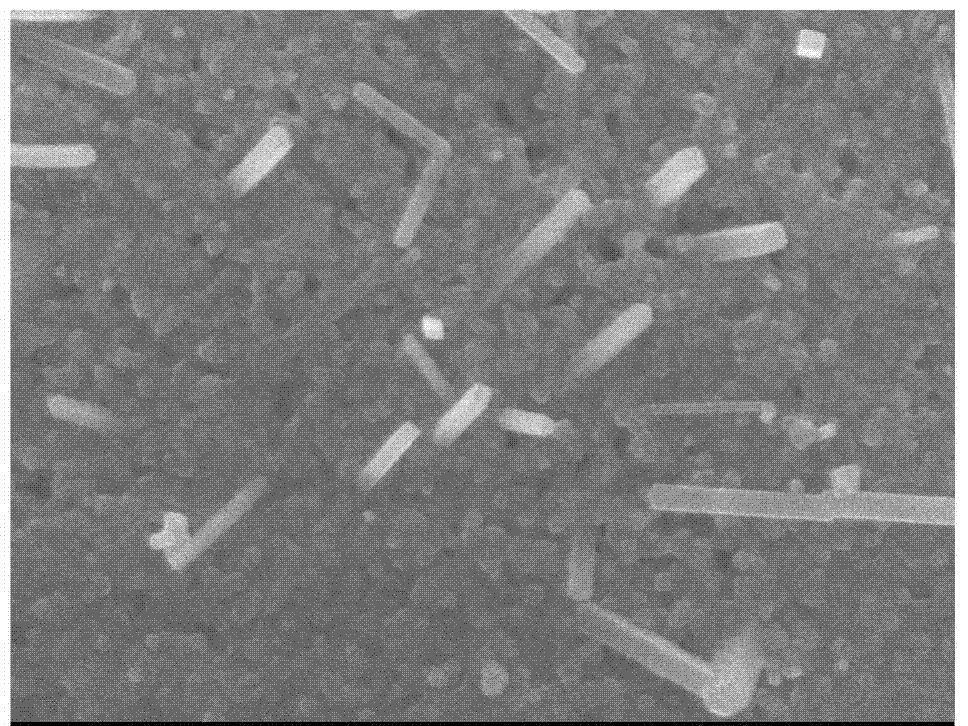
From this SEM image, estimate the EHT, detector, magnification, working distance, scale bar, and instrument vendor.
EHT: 5 kV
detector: SEI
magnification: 60 K X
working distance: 7.9 mm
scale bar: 100 nm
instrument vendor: JEOL